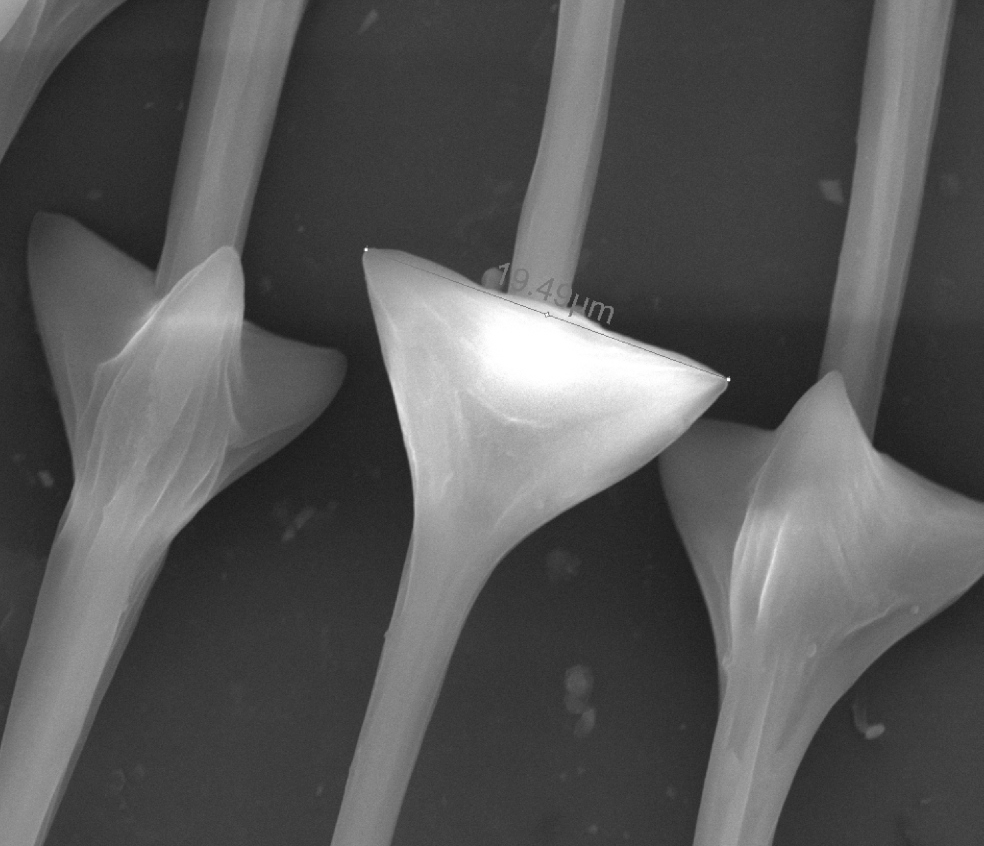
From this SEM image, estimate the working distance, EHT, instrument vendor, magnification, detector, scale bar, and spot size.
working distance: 10.8 mm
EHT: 20 kV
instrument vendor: FEI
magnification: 6 K X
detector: ETD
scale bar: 20000 nm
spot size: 4